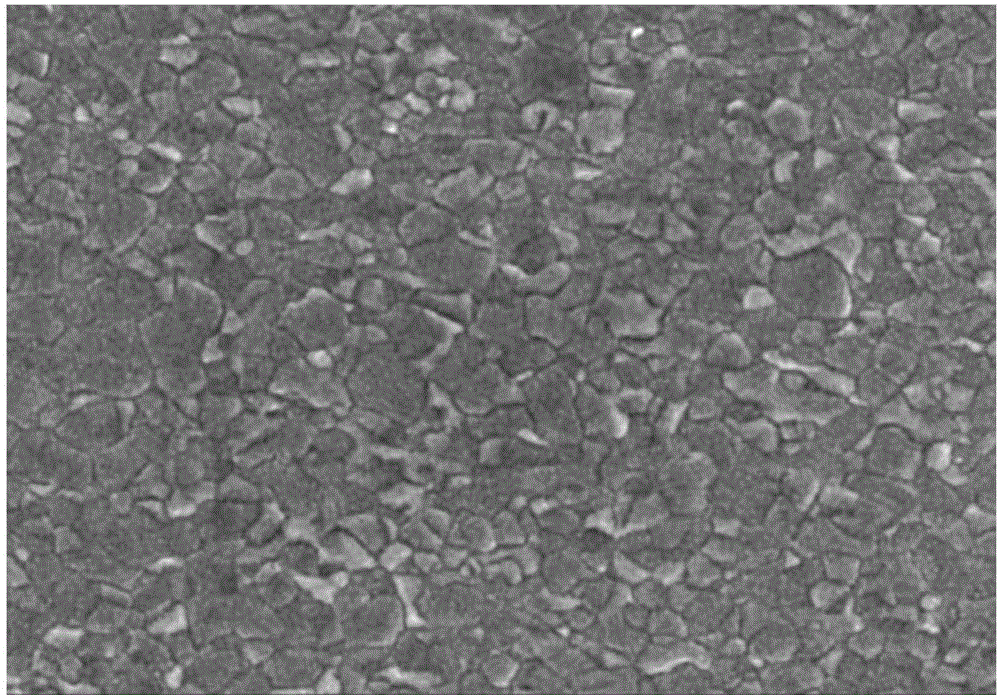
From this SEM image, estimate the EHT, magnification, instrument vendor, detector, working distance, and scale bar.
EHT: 15 kV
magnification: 4 K X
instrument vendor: Hitachi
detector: SE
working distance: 14.3 mm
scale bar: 10000 nm